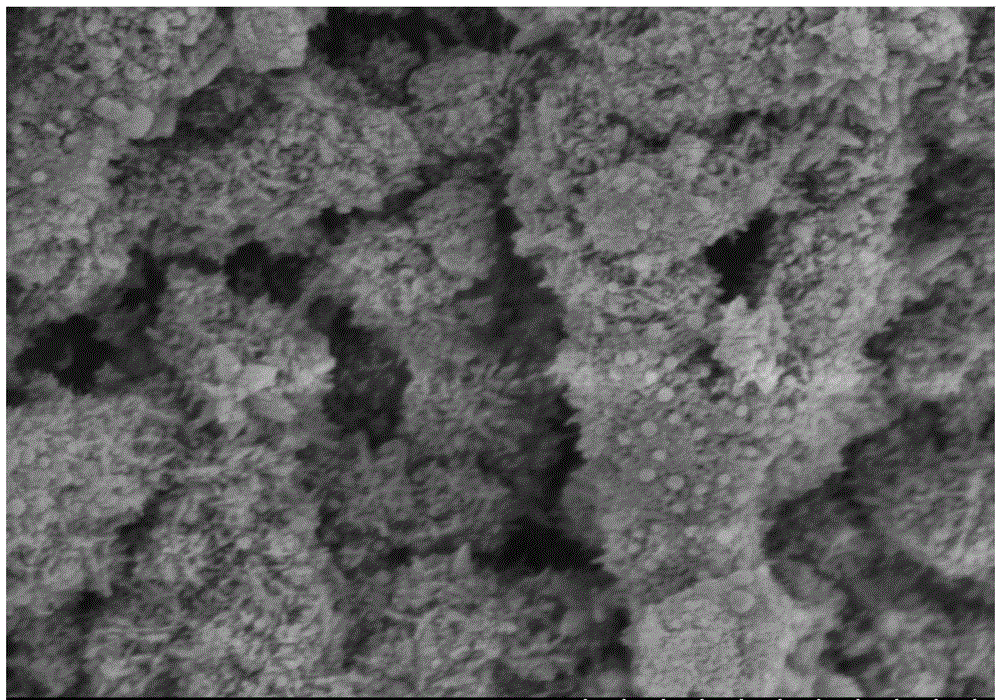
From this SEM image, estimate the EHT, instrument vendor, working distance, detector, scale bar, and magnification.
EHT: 3 kV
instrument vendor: Hitachi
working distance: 9.5 mm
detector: SE(M)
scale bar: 1000 nm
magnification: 50 K X